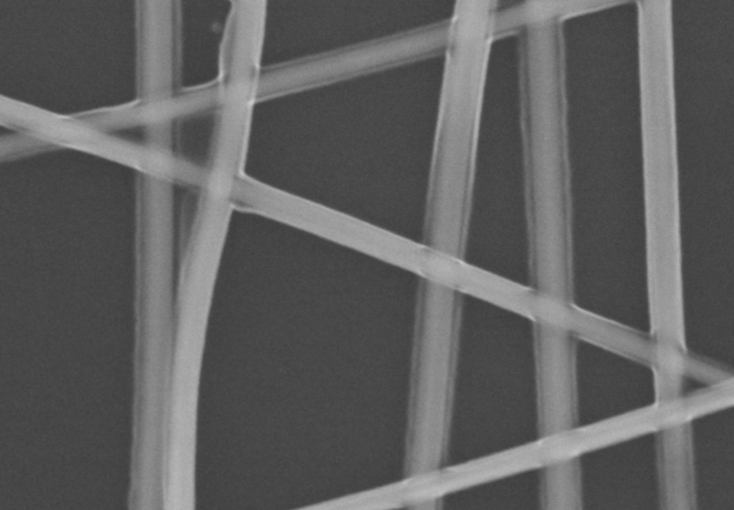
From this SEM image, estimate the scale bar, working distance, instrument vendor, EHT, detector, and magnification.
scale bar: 400 nm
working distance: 8.6 mm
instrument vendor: Hitachi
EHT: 10 kV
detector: SE(M)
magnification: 130 K X